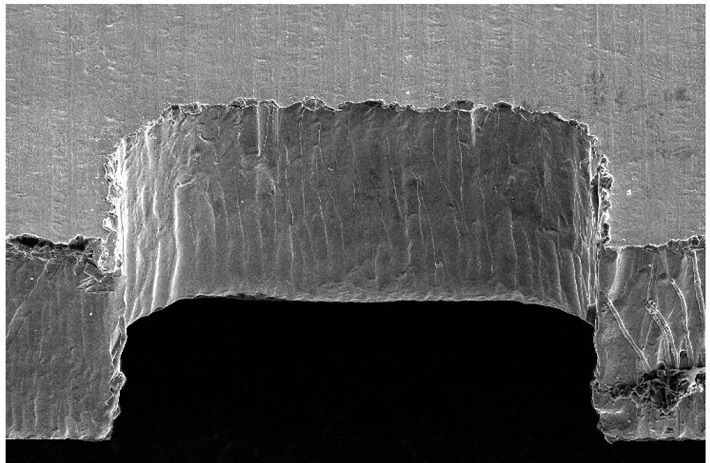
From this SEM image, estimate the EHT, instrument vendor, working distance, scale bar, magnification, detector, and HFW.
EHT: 10 kV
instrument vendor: FEI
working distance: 7.7 mm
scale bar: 100000 nm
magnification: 0.35 K X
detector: ETD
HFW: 363 µm